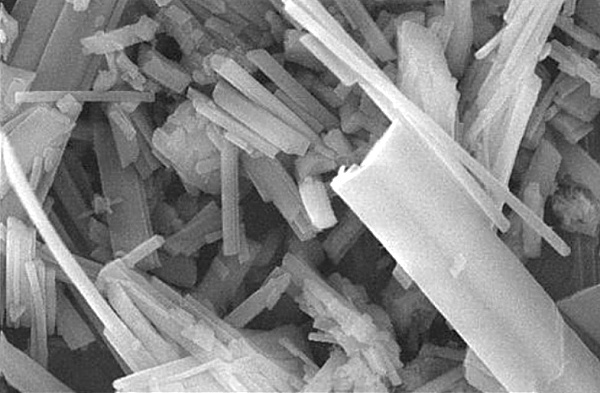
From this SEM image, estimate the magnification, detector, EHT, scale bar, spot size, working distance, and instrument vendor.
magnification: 10 K X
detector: TLD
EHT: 20 kV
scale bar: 5000 nm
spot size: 3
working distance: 5 mm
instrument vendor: FEI